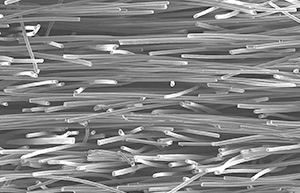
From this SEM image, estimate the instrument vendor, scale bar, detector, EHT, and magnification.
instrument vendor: JEOL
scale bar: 100000 nm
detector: SEI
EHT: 10 kV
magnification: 0.1 K X